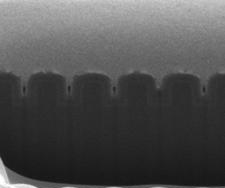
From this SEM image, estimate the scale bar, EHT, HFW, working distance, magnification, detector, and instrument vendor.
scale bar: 500 nm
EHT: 5 kV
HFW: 1.49 µm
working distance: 9.9 mm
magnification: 200 K X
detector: ETD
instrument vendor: FEI